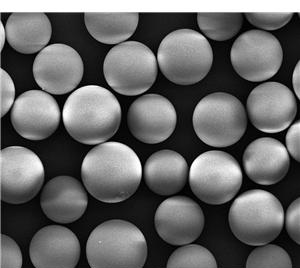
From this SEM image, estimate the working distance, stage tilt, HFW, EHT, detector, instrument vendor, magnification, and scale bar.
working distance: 8.5 mm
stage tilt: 0°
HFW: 149 µm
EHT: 20 kV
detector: ETD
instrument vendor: FEI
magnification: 2 K X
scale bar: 50000 nm